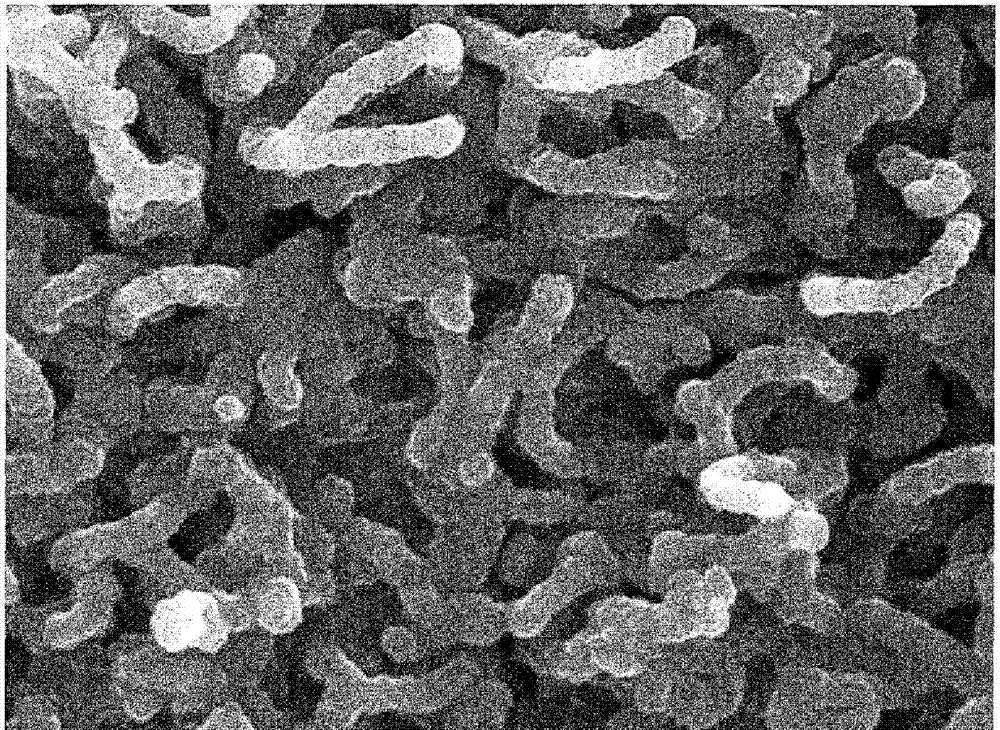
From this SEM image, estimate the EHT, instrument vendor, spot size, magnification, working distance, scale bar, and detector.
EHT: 10 kV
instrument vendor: FEI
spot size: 3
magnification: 35 K X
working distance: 5.1 mm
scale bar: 1000 nm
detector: TLD